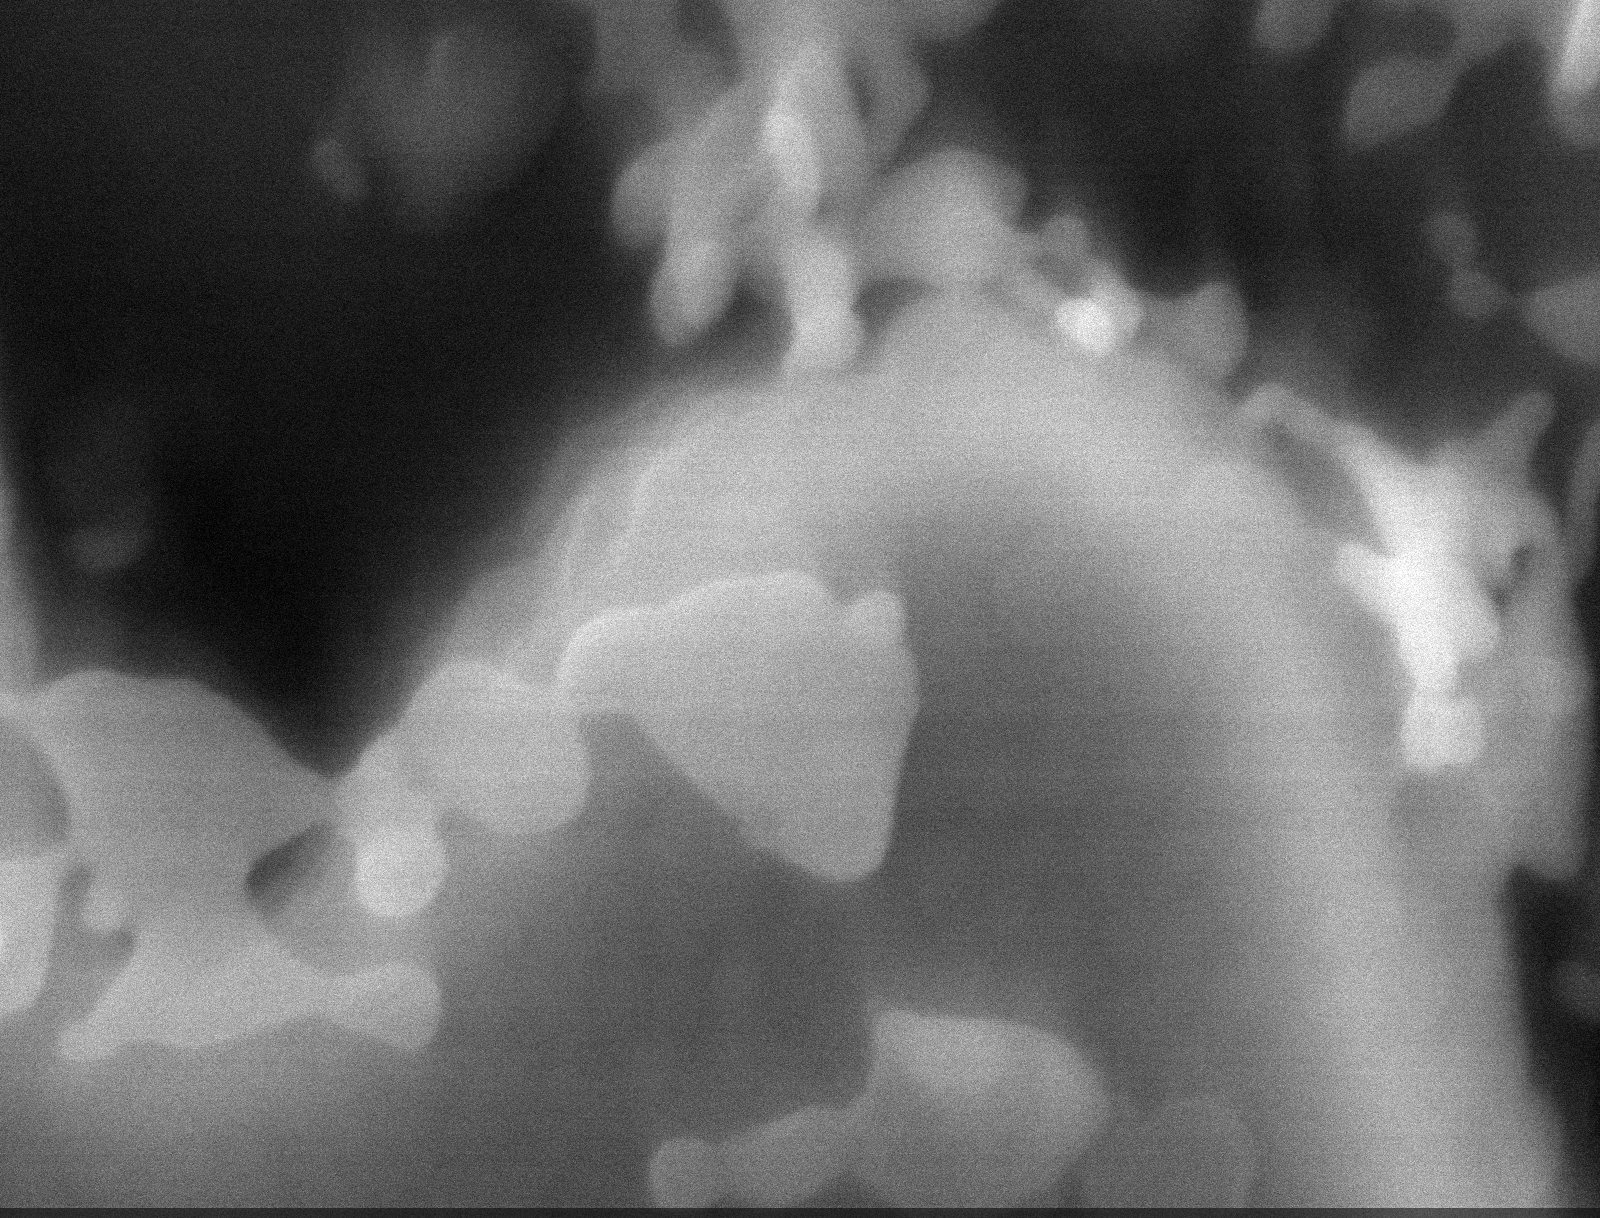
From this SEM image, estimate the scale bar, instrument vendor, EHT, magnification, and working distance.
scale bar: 200 nm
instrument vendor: Tescan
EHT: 12 kV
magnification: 100 K X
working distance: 6 mm